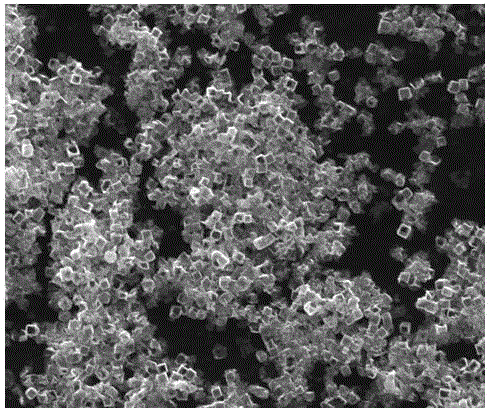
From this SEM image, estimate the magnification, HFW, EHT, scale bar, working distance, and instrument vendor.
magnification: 10 K X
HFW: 29.8 µm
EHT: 25 kV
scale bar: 10000 nm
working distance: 12.3 mm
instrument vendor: FEI